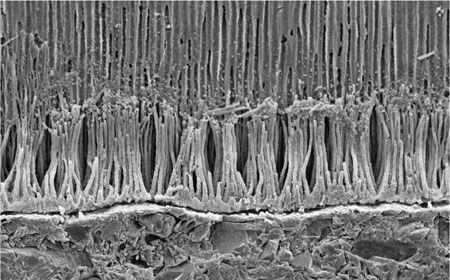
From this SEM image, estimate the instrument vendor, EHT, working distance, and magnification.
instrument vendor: other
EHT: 15 kV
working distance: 23 mm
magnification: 0.5 K X